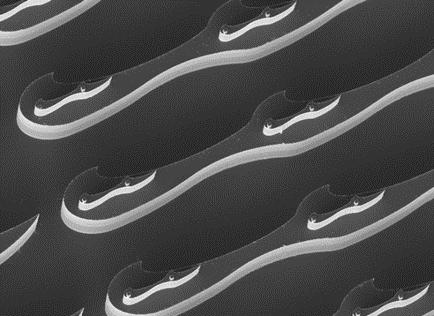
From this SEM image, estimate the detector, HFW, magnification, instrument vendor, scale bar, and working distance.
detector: SE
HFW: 519 µm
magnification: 0.4 K X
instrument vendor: Tescan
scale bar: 100000 nm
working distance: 19.9 mm